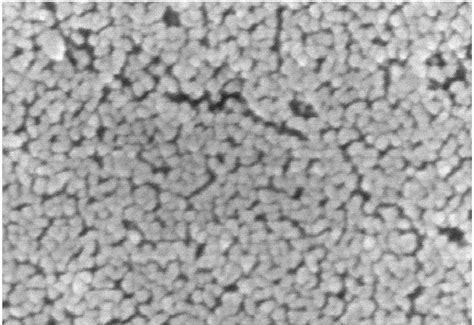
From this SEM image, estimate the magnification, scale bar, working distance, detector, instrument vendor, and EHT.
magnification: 100 K X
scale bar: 500 nm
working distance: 11 mm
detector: SE(M)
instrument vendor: Hitachi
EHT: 15 kV